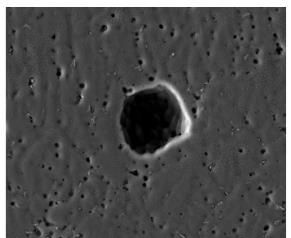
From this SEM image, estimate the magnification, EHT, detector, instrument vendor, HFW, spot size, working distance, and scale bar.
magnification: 1 K X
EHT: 30 kV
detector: ETD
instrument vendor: FEI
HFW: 256 µm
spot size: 5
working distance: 11.9 mm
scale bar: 50000 nm